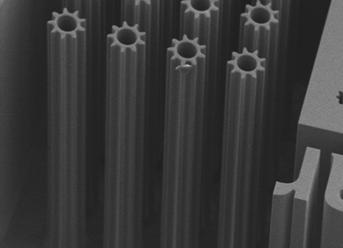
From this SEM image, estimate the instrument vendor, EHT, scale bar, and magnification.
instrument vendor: JEOL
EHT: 15 kV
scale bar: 100000 nm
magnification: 0.1 K X